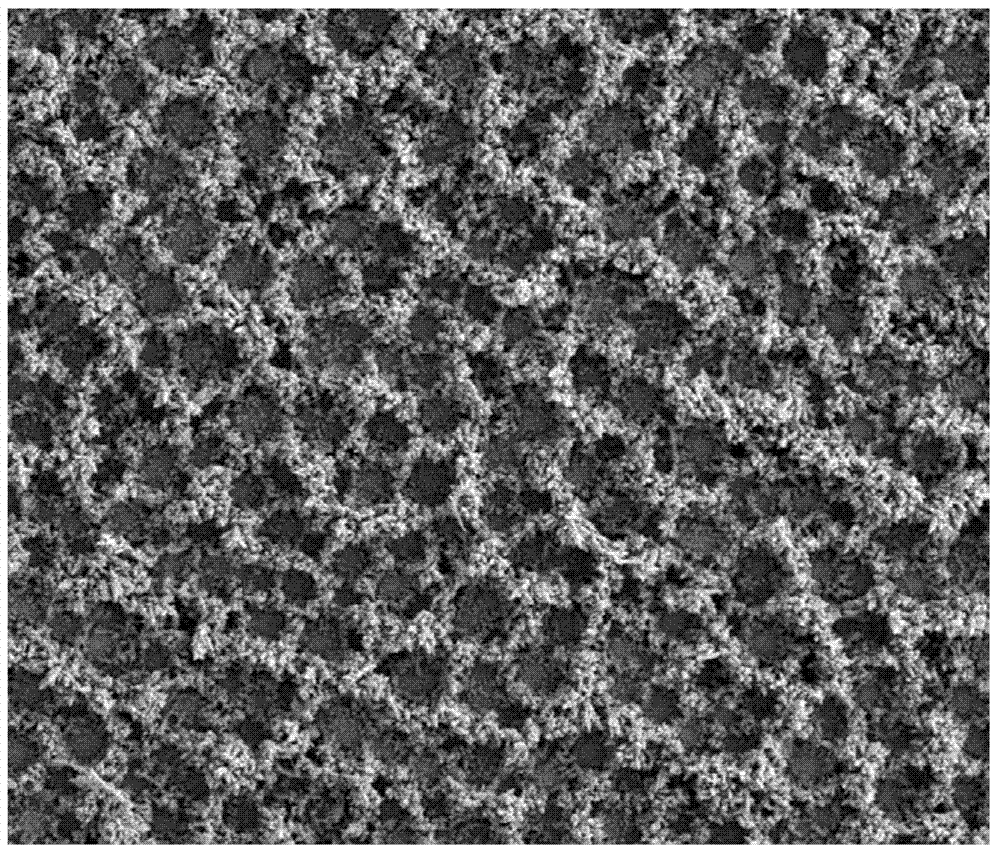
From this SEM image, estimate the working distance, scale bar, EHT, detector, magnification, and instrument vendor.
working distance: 5.3 mm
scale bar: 200000 nm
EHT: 20 kV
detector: SE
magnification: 0.4 K X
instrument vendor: FEI